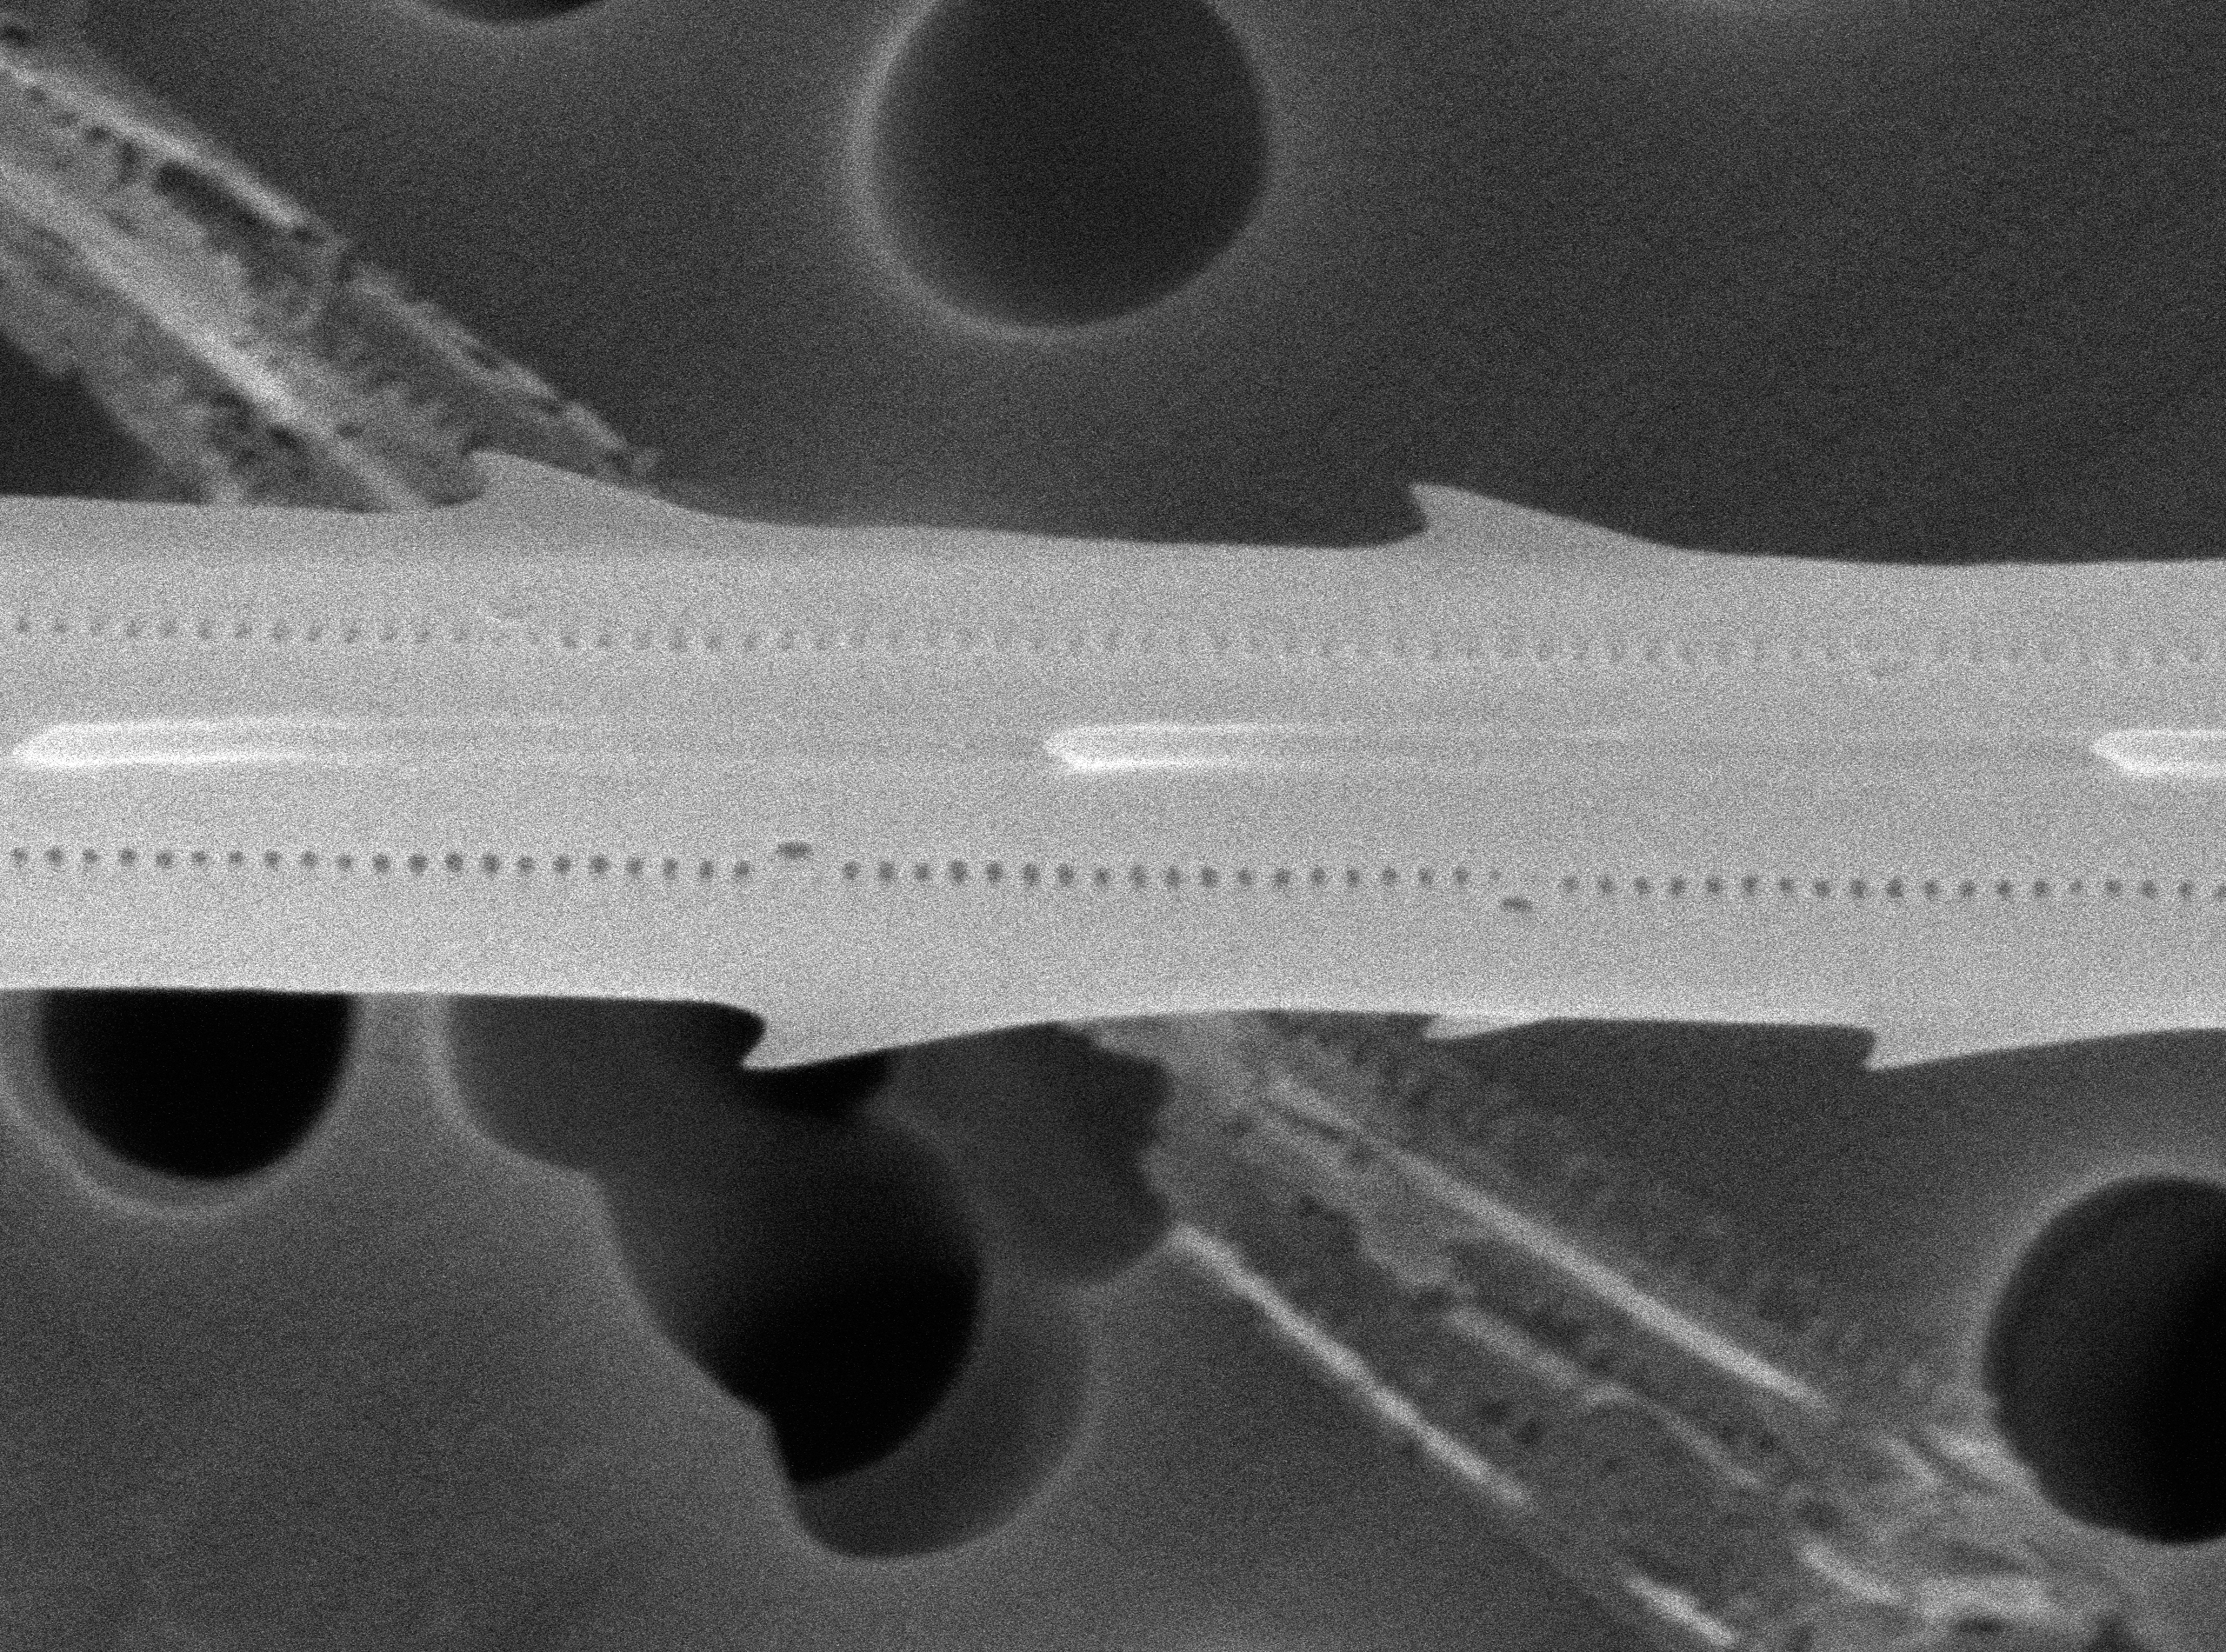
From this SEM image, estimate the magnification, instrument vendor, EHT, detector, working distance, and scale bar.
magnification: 27 K X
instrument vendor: JEOL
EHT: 5 kV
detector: SED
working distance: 10.7 mm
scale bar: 500 nm